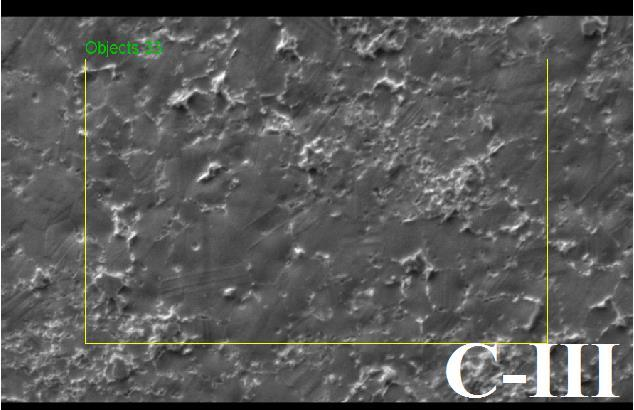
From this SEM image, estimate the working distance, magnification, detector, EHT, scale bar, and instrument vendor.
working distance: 7.8 mm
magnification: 1 K X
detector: SE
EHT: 15 kV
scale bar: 60000 nm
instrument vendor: Tescan